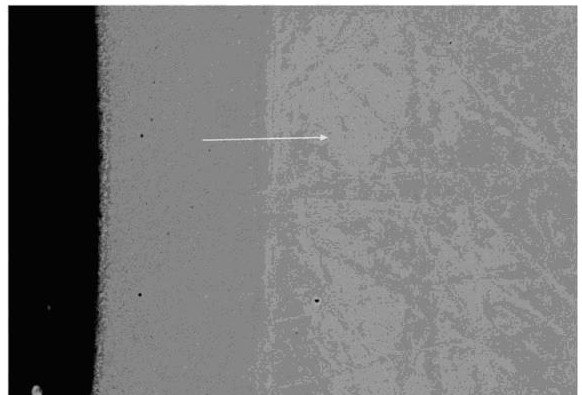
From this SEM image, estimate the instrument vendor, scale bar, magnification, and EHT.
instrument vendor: other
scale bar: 9000 nm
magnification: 2.5 K X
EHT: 15 kV